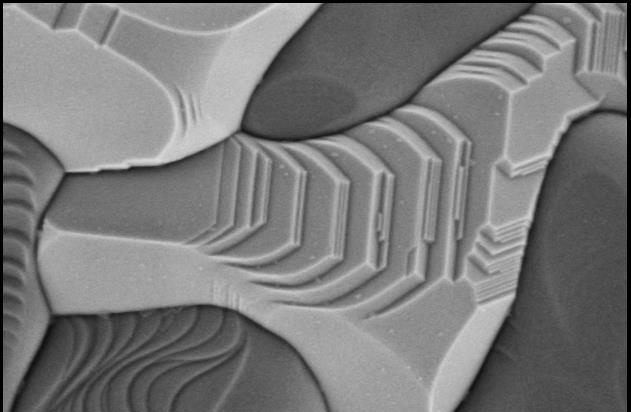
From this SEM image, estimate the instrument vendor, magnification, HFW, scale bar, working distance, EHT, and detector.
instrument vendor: Thermo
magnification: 50 K X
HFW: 2.54 µm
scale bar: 1000 nm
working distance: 3.6 mm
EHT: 1 kV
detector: T1